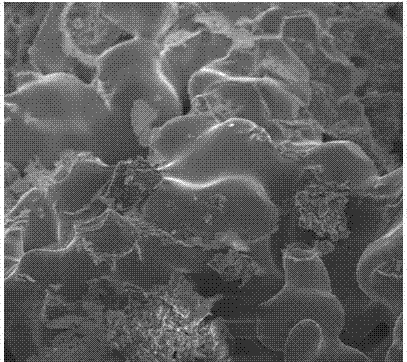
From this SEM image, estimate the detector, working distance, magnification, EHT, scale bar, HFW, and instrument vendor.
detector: TLD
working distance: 5.5 mm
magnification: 5 K X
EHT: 10 kV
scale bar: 10000 nm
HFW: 48 µm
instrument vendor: FEI